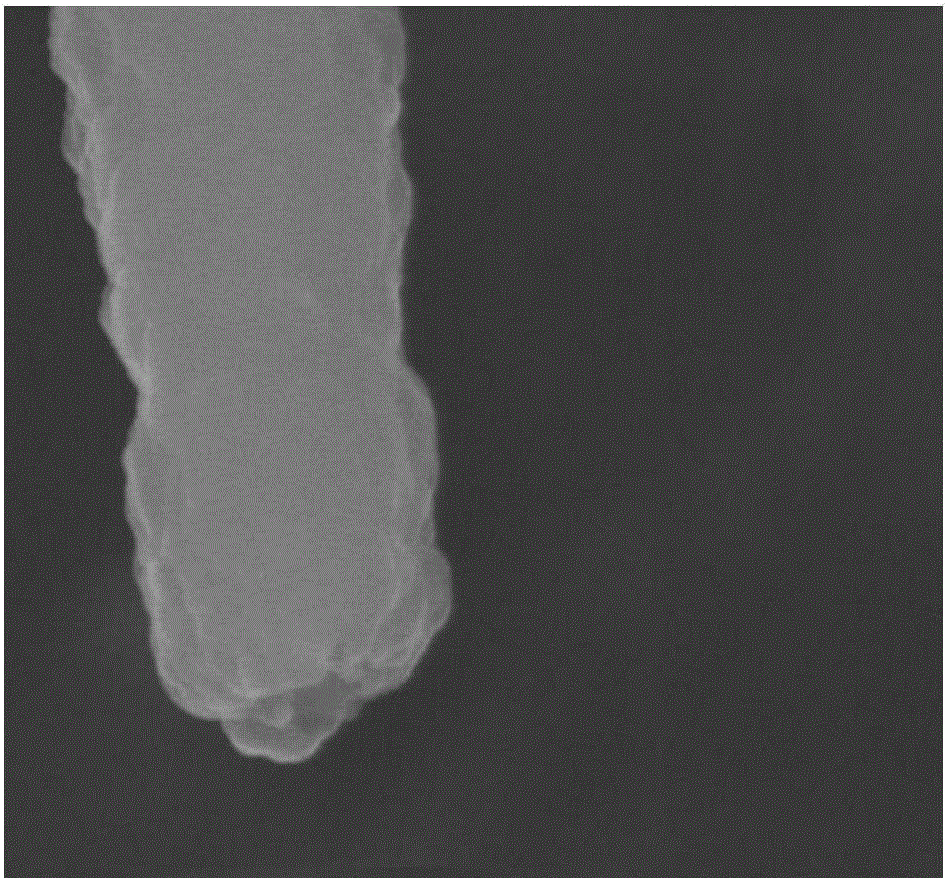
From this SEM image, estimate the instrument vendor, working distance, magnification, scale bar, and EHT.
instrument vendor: Hitachi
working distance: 5.8 mm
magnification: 100 K X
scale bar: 500 nm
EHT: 5 kV